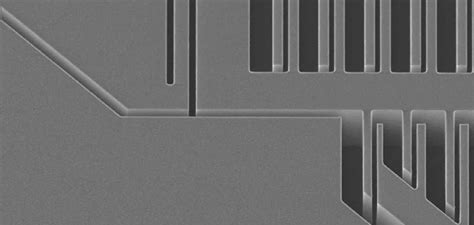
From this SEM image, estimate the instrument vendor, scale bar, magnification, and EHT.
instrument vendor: JEOL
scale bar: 50000 nm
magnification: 0.43 K X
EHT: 10 kV